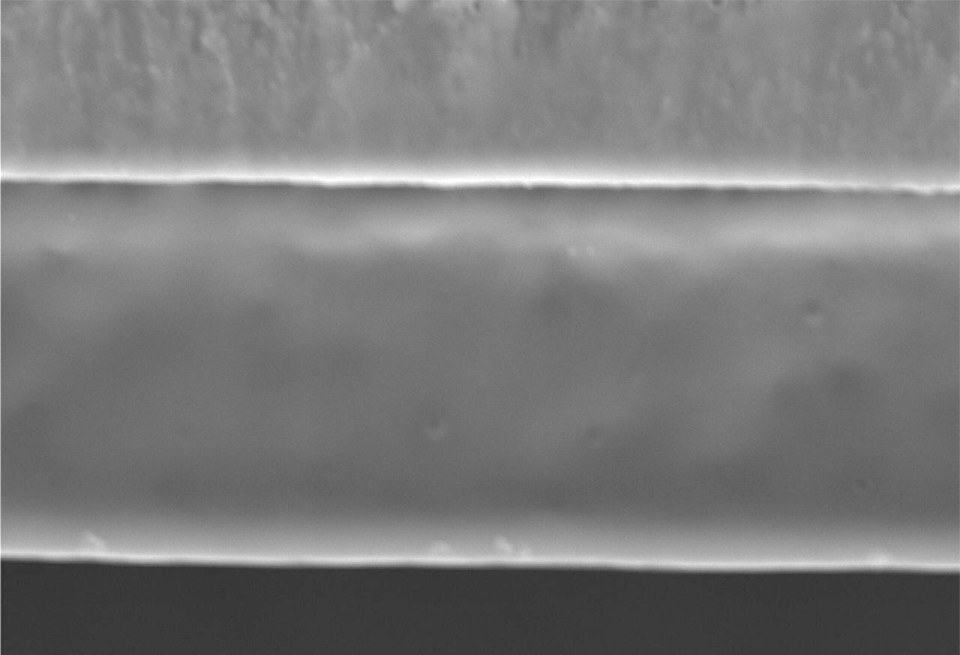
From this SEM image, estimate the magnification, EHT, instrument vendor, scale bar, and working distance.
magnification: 1.5 K X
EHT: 20 kV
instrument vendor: JEOL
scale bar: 6660 nm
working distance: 28.8 mm